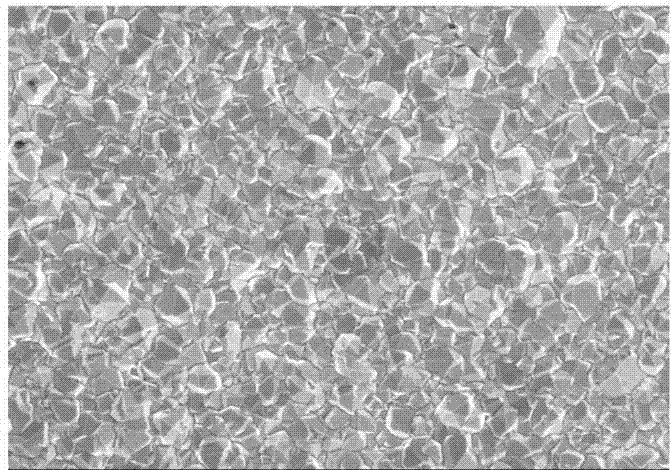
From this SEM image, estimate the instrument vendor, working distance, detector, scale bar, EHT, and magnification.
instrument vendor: Hitachi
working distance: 8.6 mm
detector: SE(M,LA0)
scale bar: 50000 nm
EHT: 15 kV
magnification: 1 K X